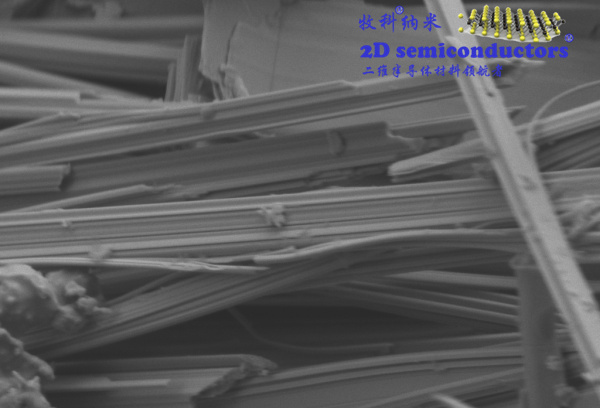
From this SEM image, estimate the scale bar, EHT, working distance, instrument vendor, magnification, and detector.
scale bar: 200 nm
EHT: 2 kV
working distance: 7 mm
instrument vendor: Zeiss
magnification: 20 K X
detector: SE2